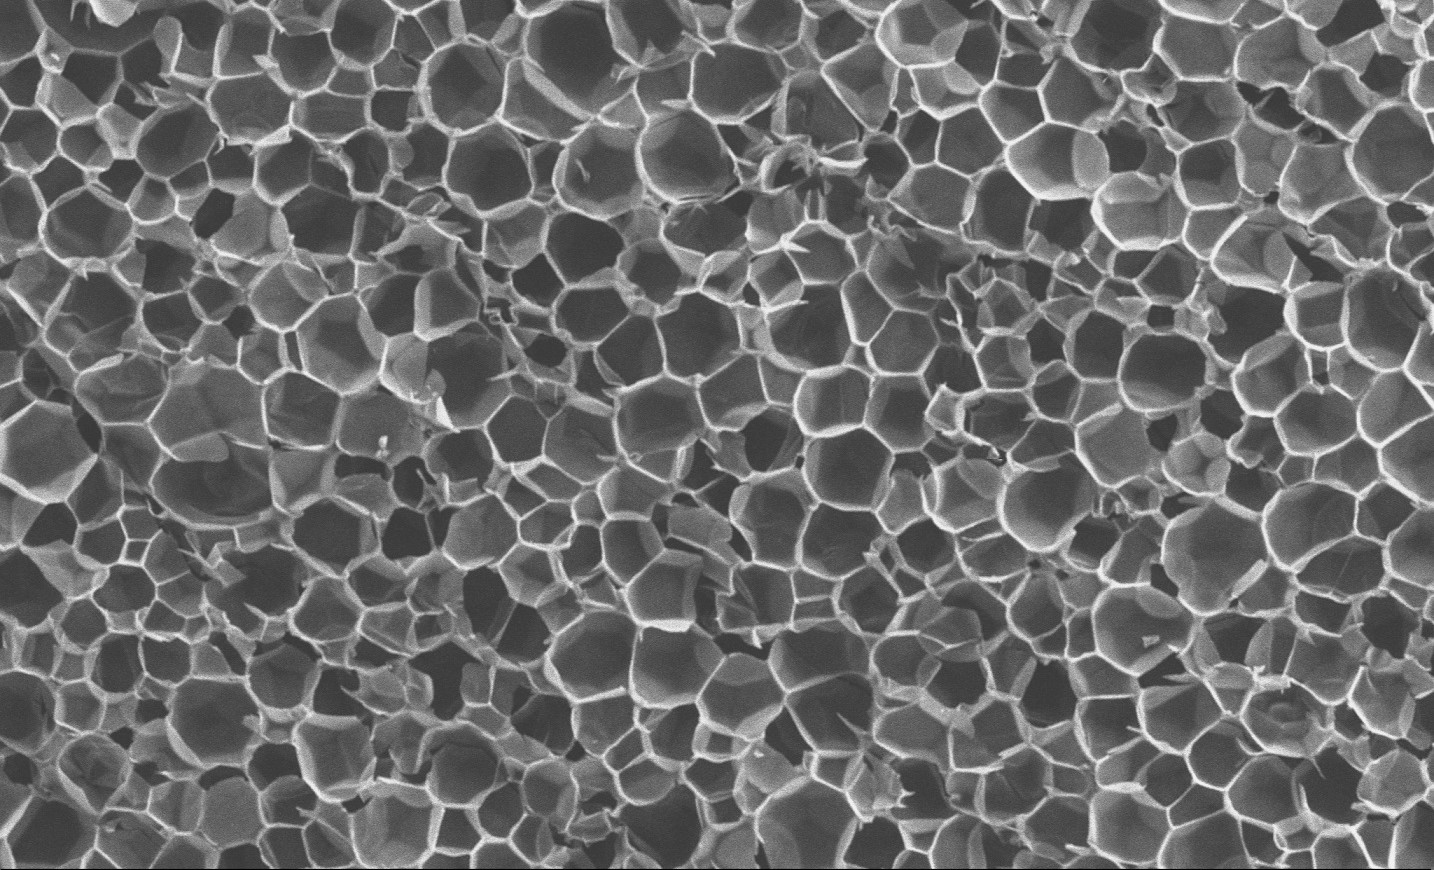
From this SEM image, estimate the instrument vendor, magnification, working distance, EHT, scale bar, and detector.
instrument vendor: Zeiss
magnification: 0.1 K X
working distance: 7.6 mm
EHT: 15 kV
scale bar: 100000 nm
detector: SE2